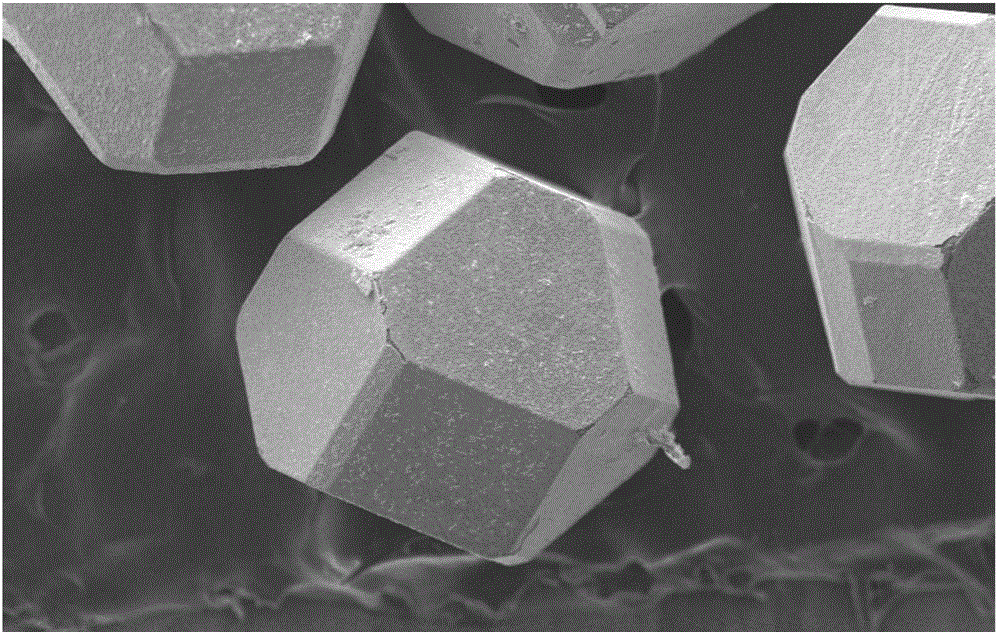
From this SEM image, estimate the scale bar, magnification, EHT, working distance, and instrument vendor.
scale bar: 100000 nm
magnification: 0.325 K X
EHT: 15 kV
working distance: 11 mm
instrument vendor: Zeiss